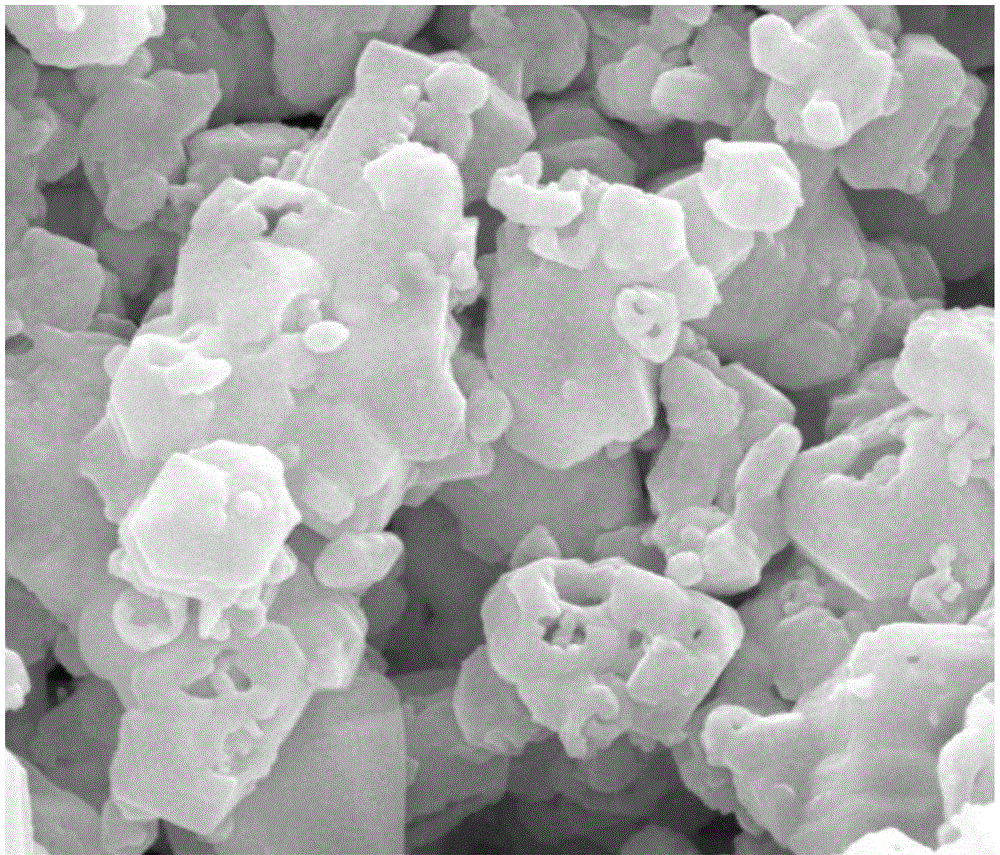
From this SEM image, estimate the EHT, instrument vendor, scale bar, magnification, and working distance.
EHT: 20 kV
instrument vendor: FEI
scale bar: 1000 nm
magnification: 100 K X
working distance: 4.4 mm